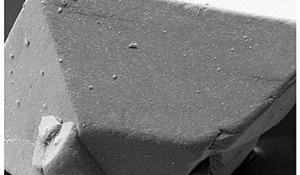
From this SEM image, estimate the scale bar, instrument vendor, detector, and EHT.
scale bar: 20000 nm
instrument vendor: Zeiss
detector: SE1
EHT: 10 kV